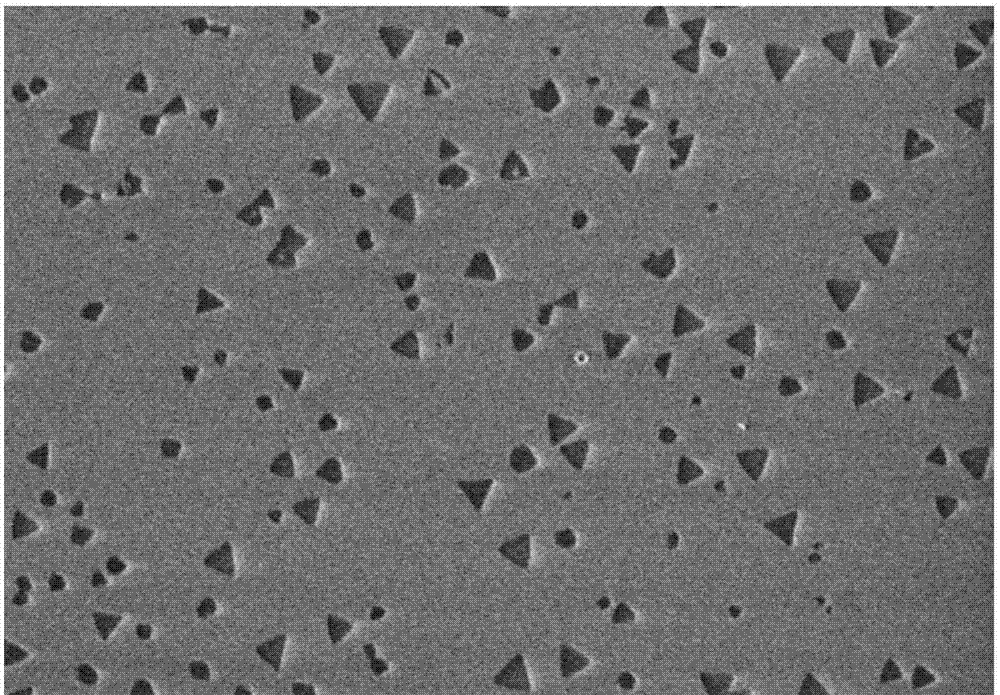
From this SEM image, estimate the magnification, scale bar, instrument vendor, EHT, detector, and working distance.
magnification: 6 K X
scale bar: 5000 nm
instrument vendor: Hitachi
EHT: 1 kV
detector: SE(U)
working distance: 9.7 mm